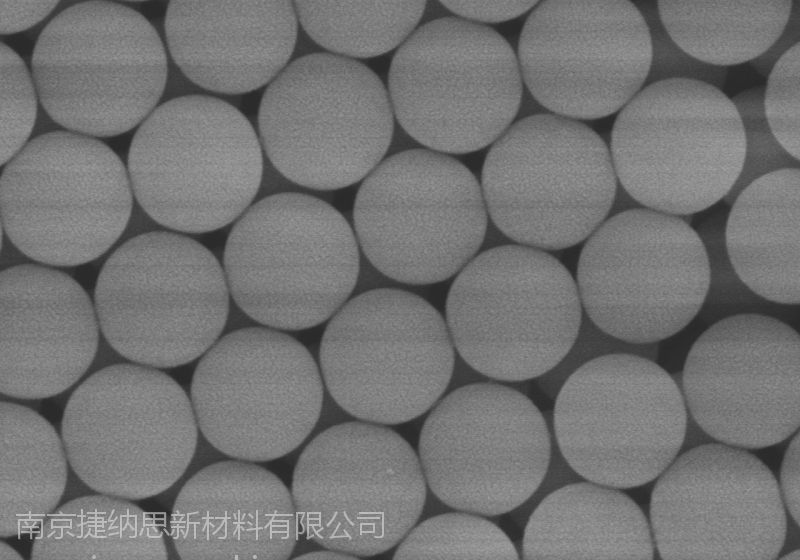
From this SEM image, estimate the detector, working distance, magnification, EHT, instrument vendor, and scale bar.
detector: SE(M)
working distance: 8.4 mm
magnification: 50 K X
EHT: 5 kV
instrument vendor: Hitachi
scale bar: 1000 nm